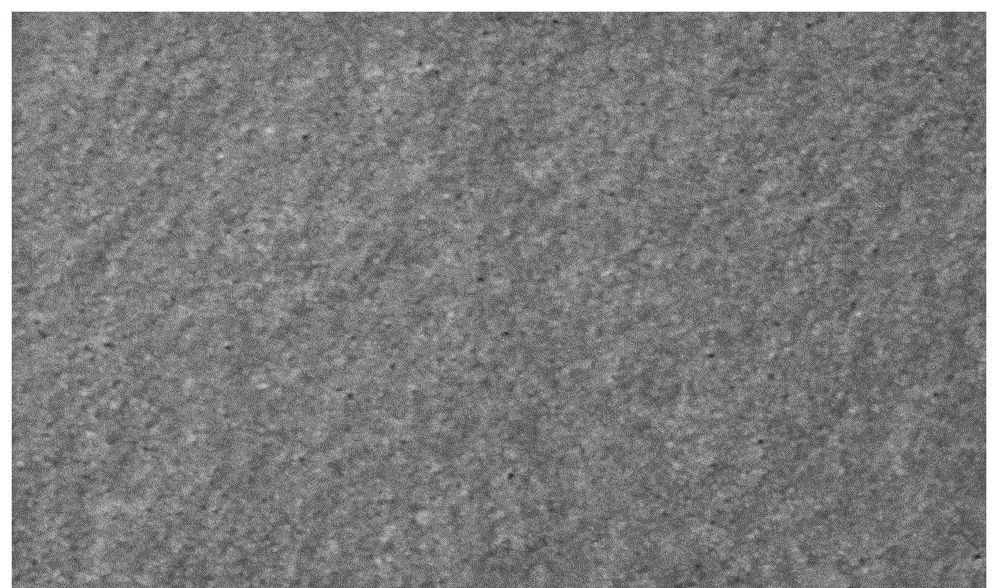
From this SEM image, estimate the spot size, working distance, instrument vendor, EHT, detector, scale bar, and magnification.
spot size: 3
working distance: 7.3 mm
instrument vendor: FEI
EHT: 15 kV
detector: SE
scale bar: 500 nm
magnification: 40 K X